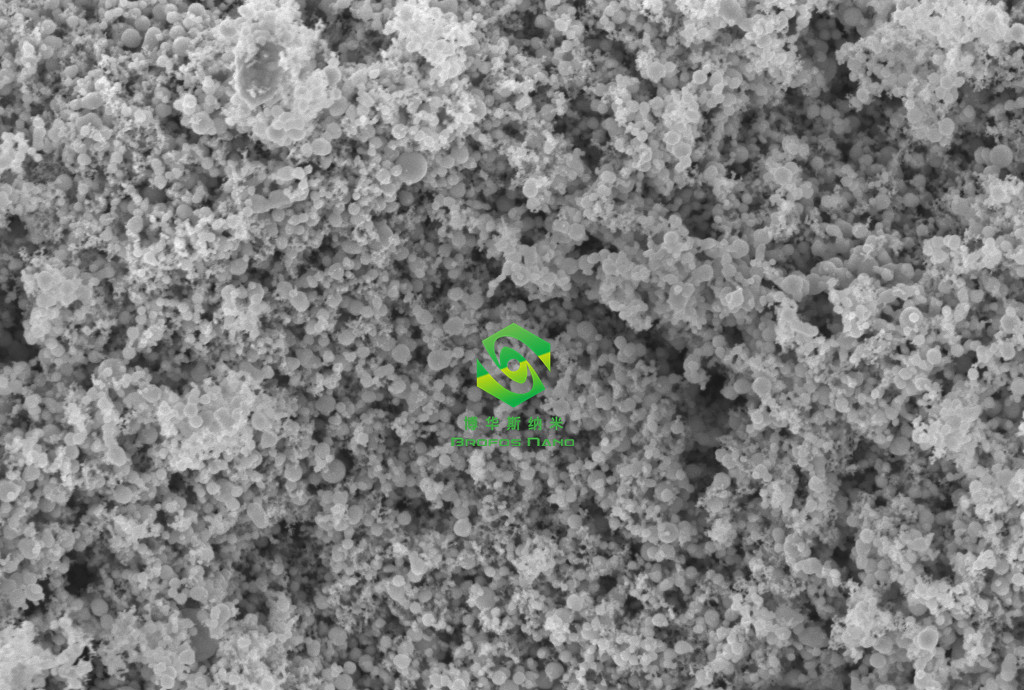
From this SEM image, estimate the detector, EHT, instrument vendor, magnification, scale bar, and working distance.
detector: InLens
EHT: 5 kV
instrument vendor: Zeiss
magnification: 20 K X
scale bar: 200 nm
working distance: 4.2 mm